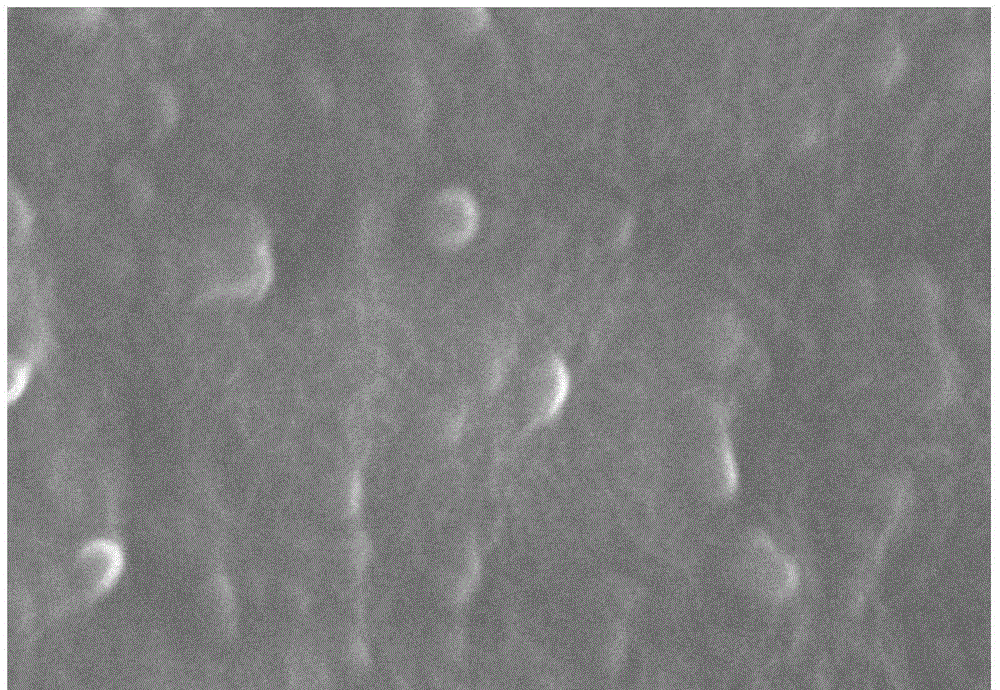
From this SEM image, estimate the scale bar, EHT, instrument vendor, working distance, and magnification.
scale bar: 500 nm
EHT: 20 kV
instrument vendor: Hitachi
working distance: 12.3 mm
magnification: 60 K X